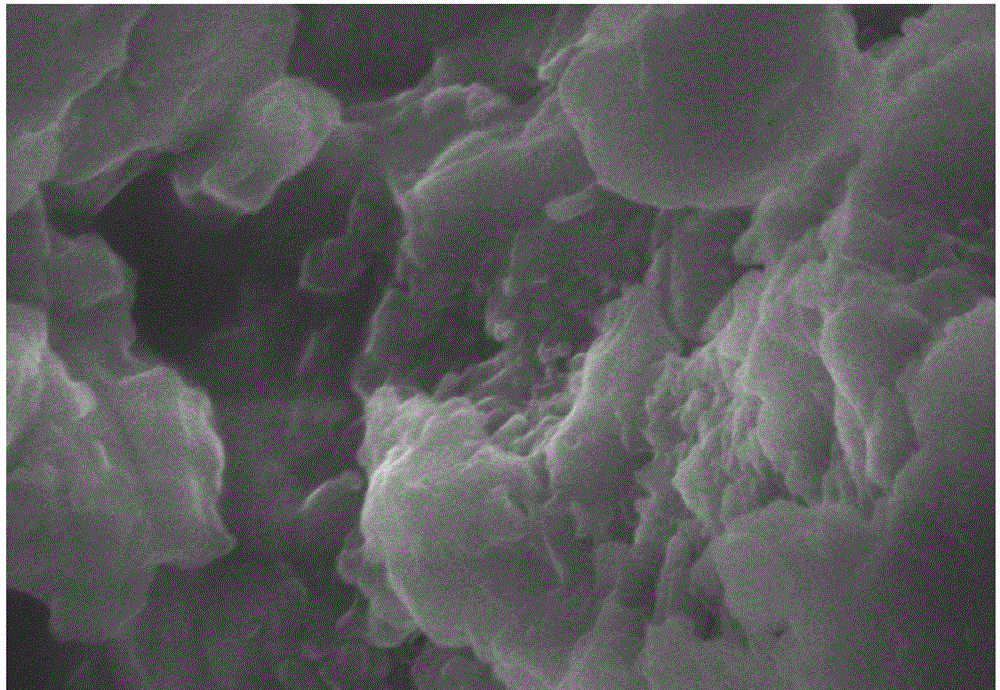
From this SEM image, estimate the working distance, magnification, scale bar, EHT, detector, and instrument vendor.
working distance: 8.1 mm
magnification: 50 K X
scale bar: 1000 nm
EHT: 5 kV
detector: SE(M)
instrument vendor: Hitachi